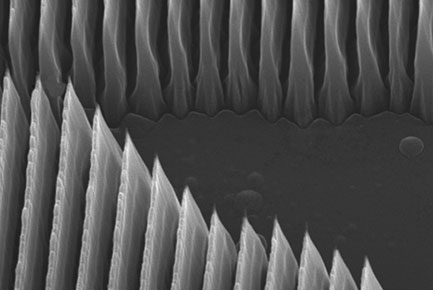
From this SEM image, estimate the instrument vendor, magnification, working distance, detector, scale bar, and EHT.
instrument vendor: JEOL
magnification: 23 K X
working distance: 10 mm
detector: SED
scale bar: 1000 nm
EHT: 15 kV